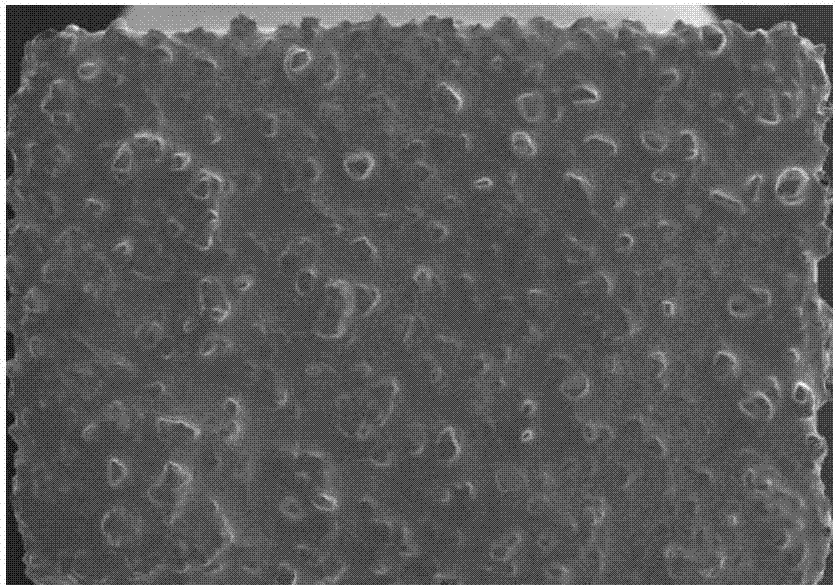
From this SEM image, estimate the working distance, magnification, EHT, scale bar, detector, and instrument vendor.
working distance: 33.7 mm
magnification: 0.025 K X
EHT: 20 kV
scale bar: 2000000 nm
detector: SE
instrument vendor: Hitachi